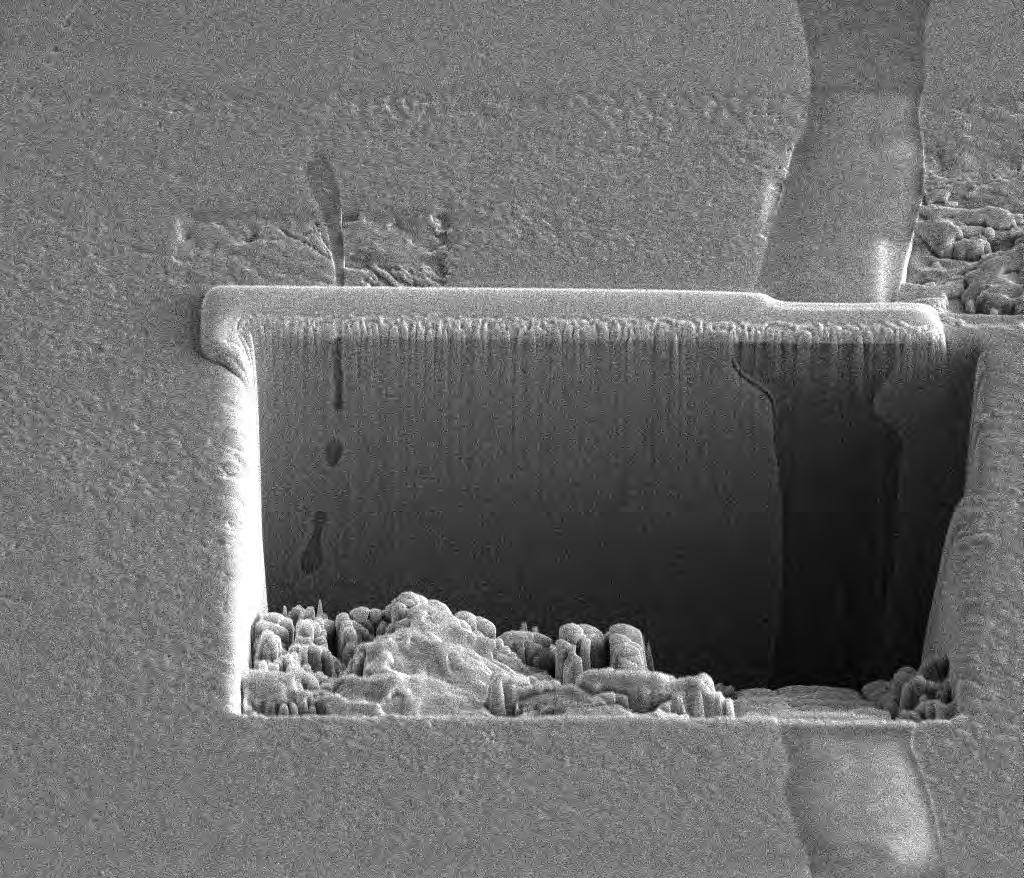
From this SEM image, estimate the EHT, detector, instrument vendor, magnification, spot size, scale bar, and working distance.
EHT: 10 kV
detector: ETD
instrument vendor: FEI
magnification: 3.5 K X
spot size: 4.5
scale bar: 20000 nm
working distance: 10 mm